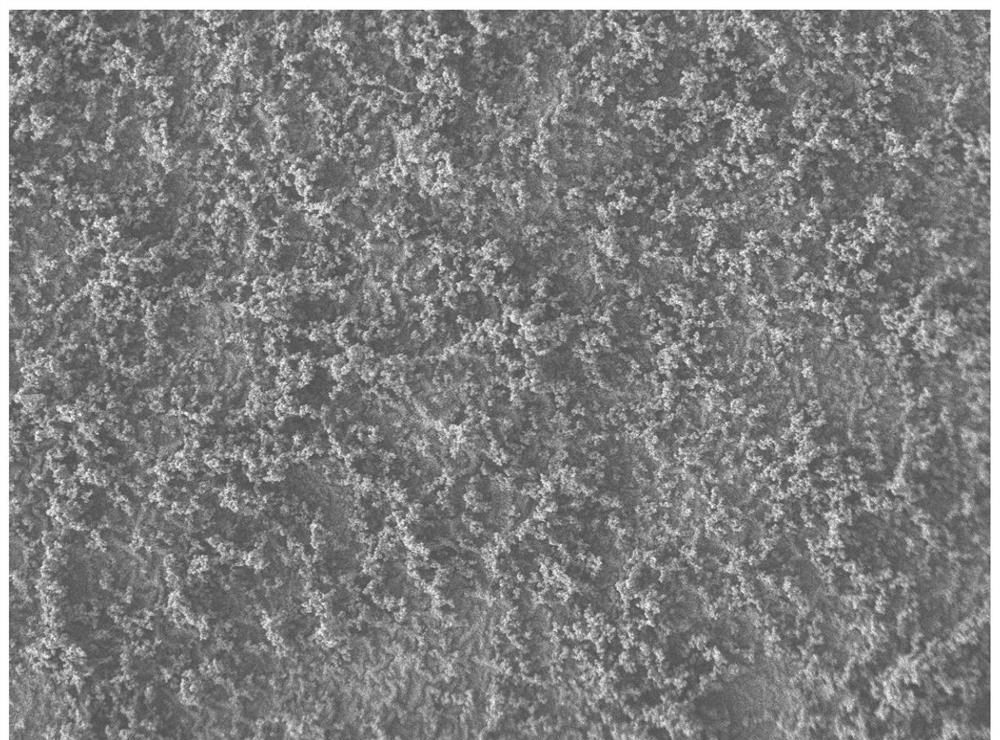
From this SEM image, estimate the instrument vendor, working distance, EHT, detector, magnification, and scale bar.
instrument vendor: JEOL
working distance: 7.9 mm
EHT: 5 kV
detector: LEI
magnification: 0.3 K X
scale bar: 10000 nm